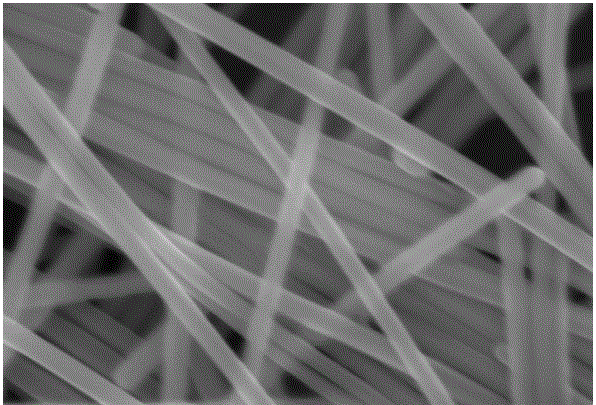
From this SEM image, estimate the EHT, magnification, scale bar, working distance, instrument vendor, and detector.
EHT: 8 kV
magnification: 100 K X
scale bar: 100 nm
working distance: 5.8 mm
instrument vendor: Zeiss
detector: InLens